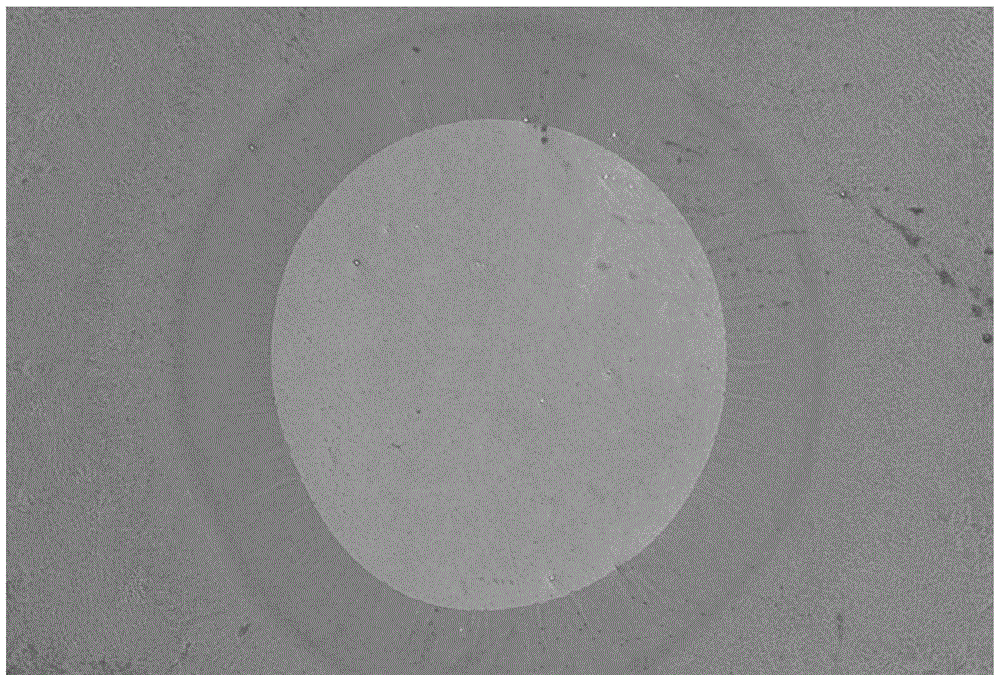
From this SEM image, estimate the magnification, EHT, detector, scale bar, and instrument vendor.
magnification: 0.27 K X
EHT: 20 kV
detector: SEI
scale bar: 50000 nm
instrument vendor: JEOL